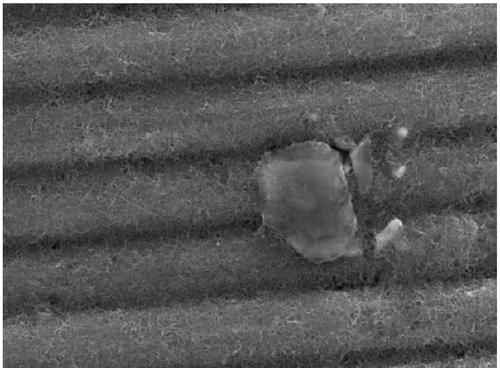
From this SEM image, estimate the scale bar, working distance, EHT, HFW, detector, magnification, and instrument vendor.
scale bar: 10000 nm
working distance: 28.64 mm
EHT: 20 kV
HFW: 55.4 µm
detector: SE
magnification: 6.25 K X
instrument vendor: Tescan